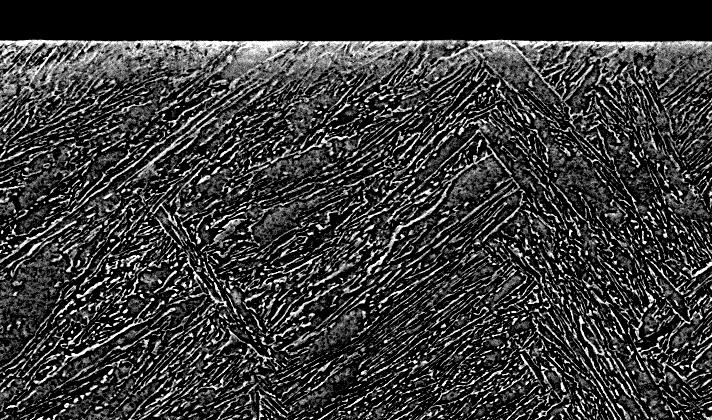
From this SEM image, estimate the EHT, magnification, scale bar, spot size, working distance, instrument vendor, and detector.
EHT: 20 kV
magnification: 1.5 K X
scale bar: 20000 nm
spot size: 5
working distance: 11.3 mm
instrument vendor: FEI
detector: BSE